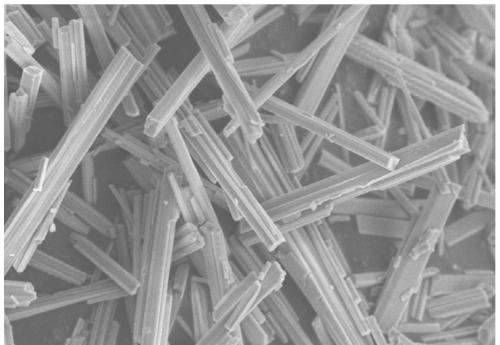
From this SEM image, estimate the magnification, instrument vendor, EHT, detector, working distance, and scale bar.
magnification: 4 K X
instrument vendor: Hitachi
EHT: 5 kV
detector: SE(M)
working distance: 9.2 mm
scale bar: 10000 nm